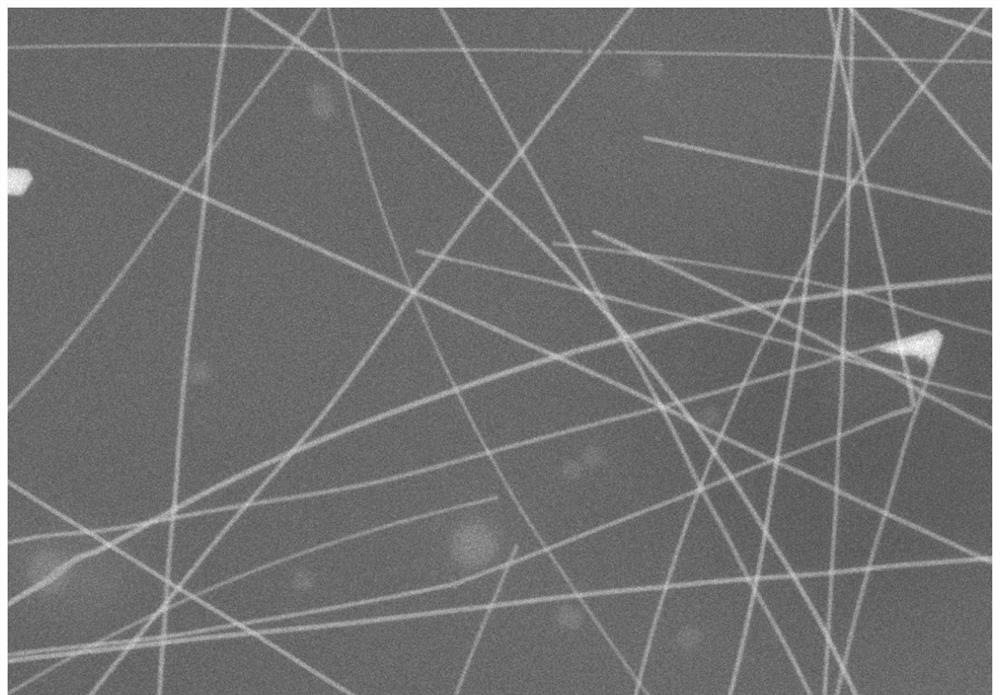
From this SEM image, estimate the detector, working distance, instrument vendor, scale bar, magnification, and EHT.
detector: LA100(U)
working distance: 8.2 mm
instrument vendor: Hitachi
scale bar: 2000 nm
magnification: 20 K X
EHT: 10 kV